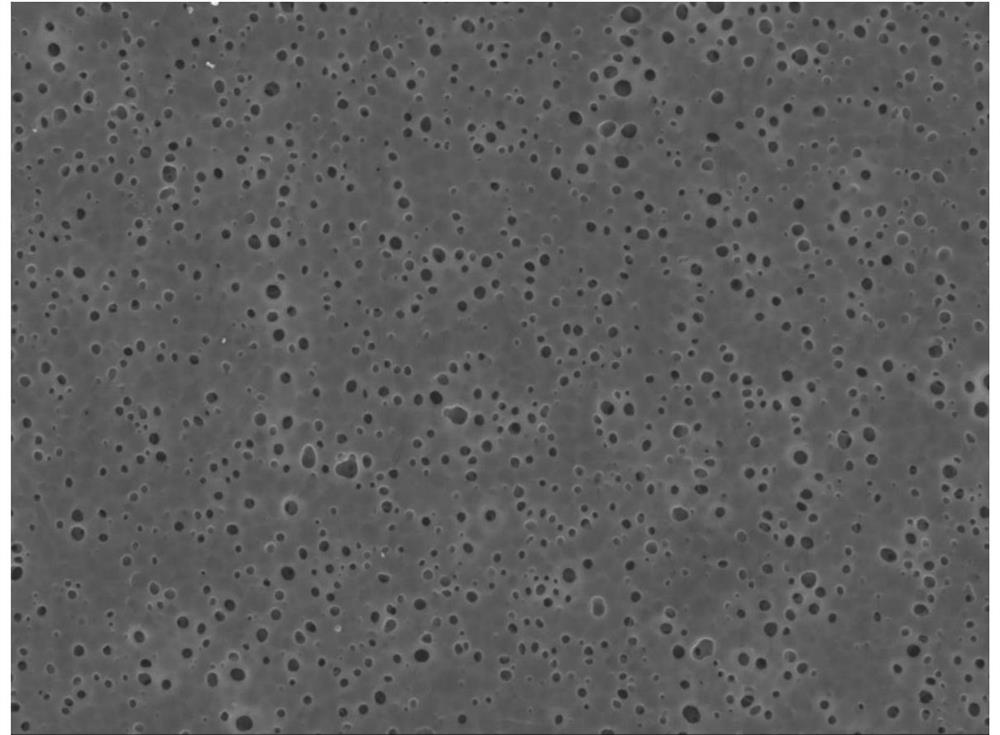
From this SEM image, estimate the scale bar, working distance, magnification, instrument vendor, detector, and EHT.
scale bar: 10000 nm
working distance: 10.7 mm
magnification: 2 K X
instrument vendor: JEOL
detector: LED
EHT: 10 kV